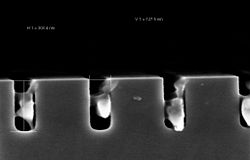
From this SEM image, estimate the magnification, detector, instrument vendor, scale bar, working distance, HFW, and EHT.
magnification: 200 K X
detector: InLens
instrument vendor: Zeiss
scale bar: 200 nm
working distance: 3.2 mm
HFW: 1.551 µm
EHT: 2 kV